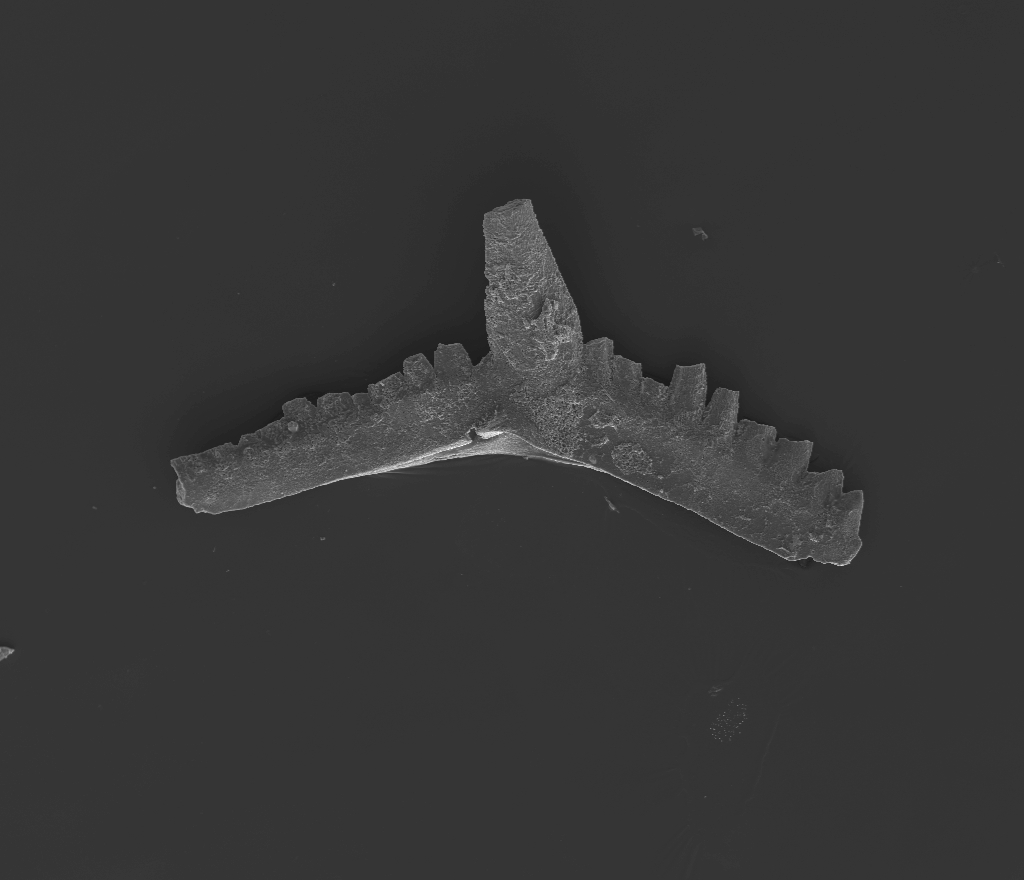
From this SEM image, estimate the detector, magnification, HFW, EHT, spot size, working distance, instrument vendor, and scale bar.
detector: ETD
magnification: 0.18 K X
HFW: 1500 µm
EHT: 20 kV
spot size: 5.5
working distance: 10 mm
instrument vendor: FEI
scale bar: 300000 nm